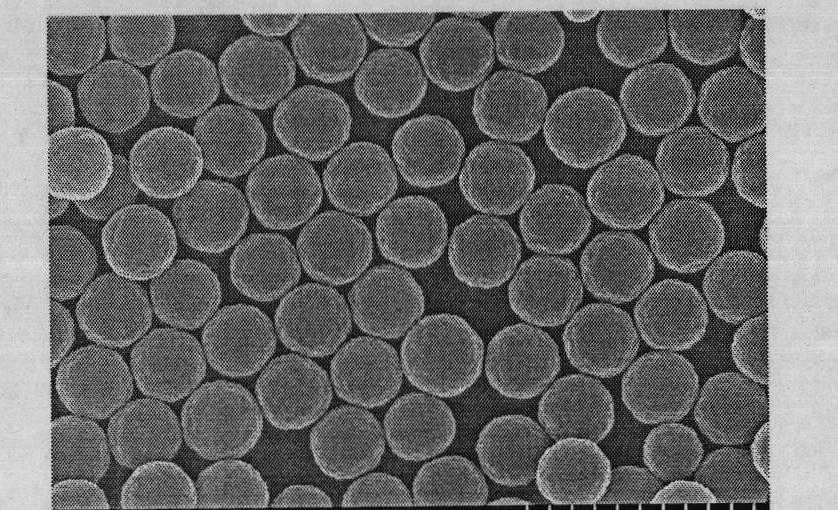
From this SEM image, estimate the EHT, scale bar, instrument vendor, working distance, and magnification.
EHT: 20 kV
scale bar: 2000 nm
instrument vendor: Hitachi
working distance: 12.1 mm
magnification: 20 K X